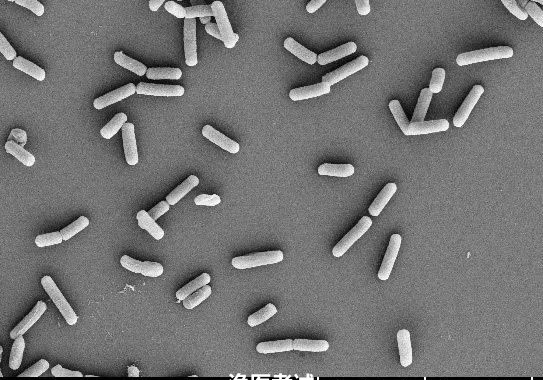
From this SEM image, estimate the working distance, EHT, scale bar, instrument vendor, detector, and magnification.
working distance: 14.3 mm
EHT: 5 kV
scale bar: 10000 nm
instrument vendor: Hitachi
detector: SE(U)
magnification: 5 K X